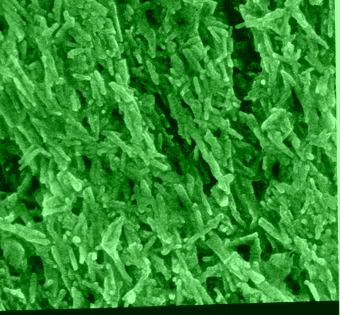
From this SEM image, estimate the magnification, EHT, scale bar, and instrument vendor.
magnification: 10 K X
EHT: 15 kV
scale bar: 1000 nm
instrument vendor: JEOL